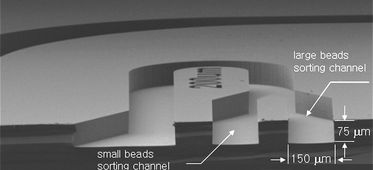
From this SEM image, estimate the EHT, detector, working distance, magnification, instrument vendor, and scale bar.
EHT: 3 kV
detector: LEI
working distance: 10 mm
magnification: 0.095 K X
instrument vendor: JEOL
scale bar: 100000 nm